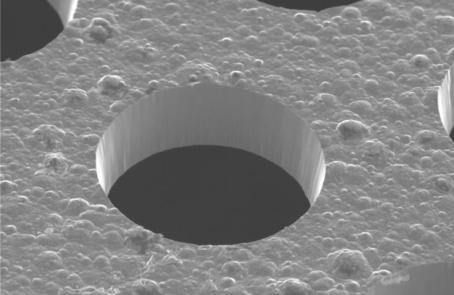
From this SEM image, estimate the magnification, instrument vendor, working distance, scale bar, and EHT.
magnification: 0.5 K X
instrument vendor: Hitachi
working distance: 20.5 mm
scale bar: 20000 nm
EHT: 20 kV